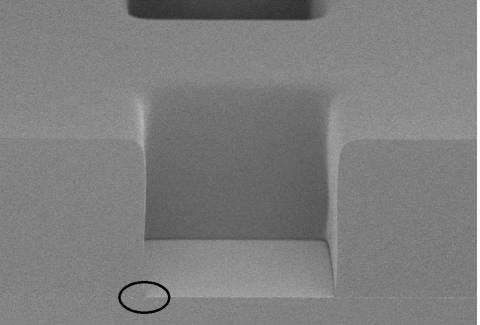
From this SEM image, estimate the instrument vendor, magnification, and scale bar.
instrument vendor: Hitachi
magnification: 1 K X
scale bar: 50000 nm